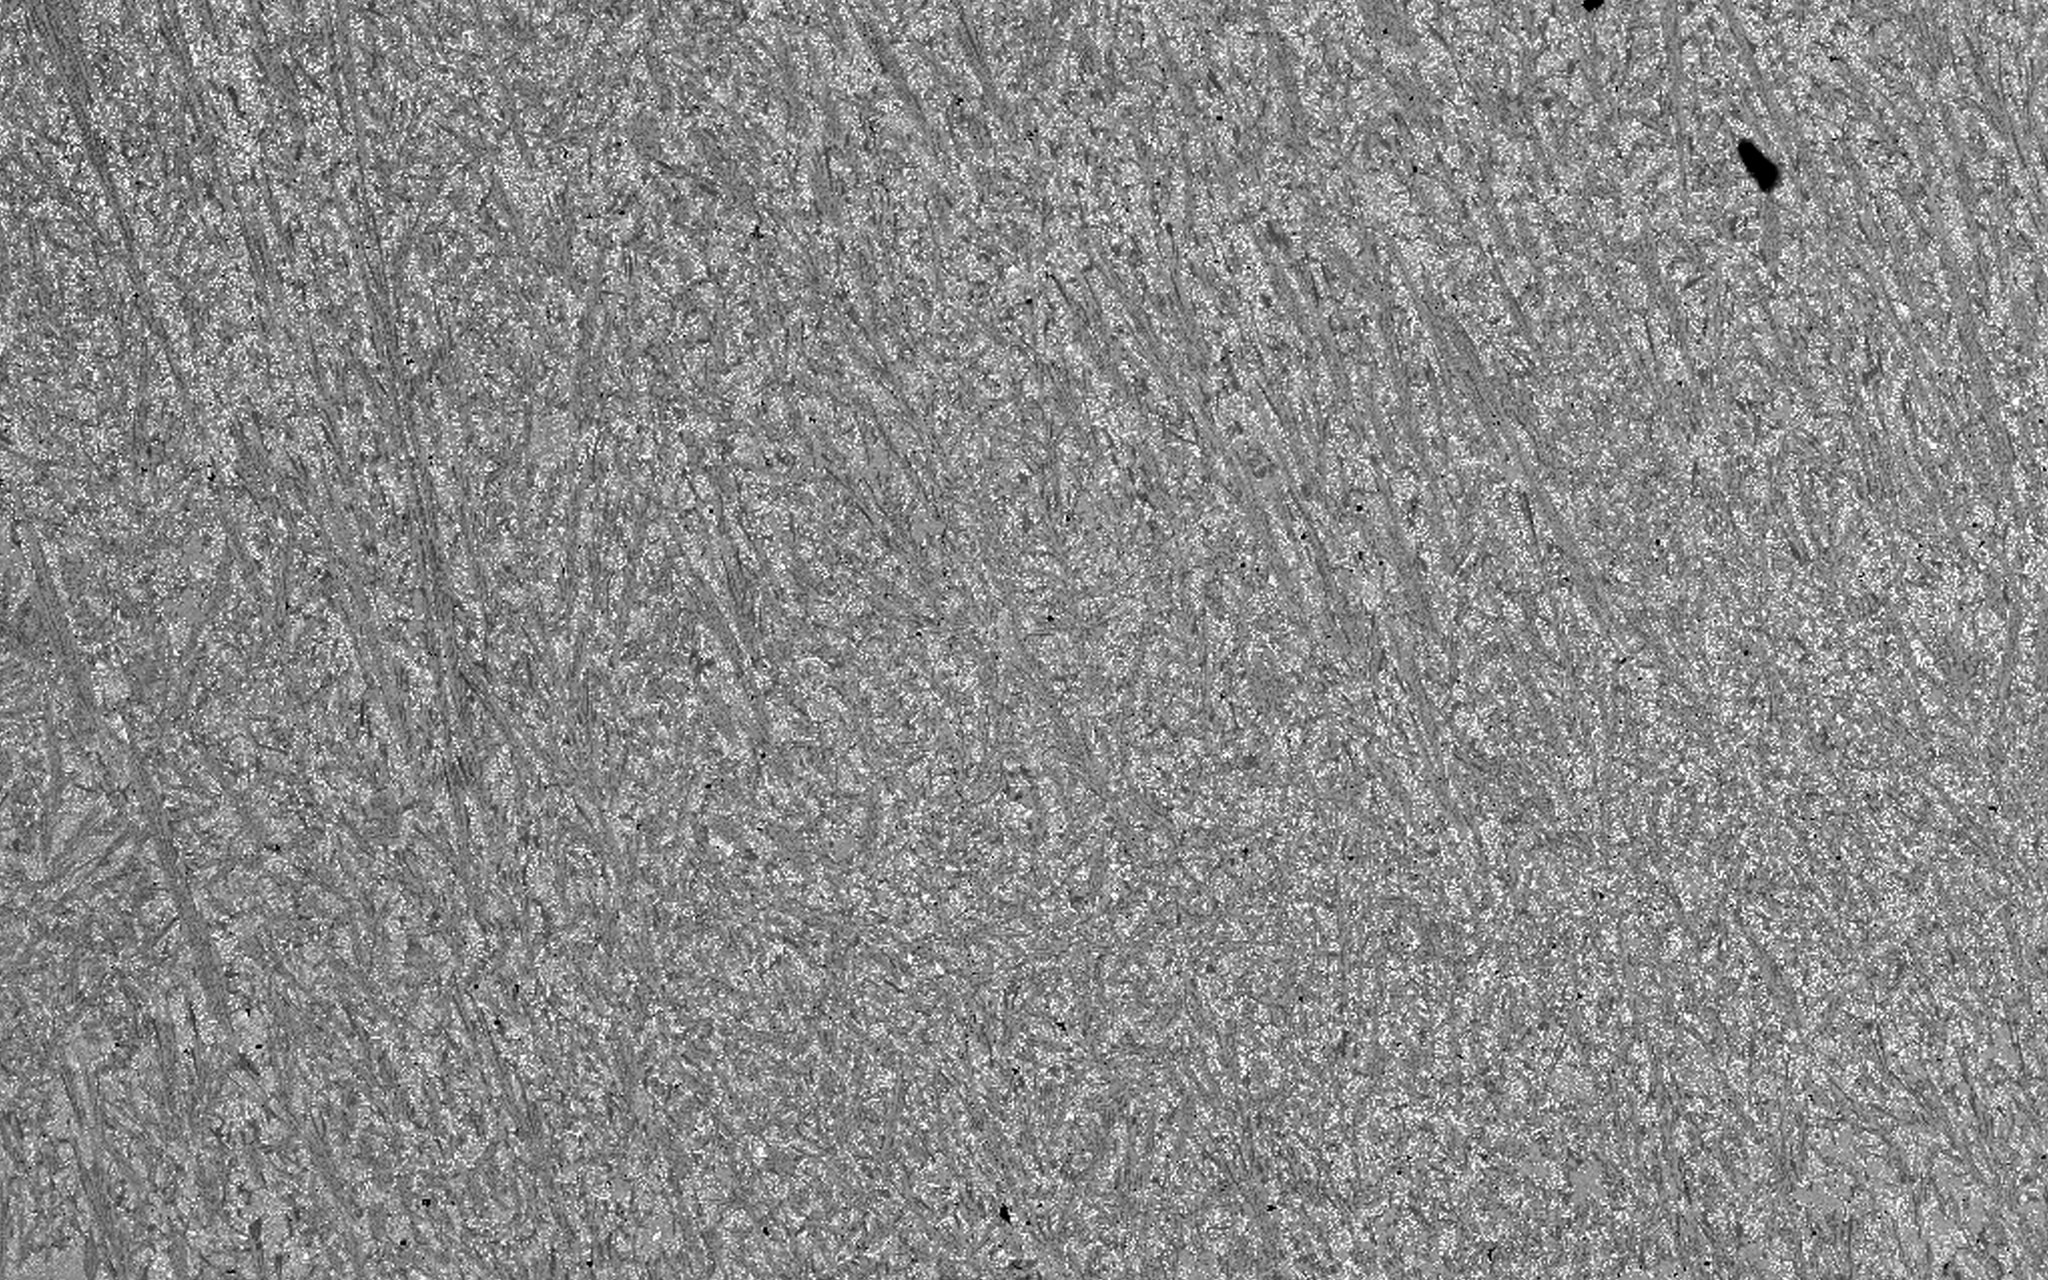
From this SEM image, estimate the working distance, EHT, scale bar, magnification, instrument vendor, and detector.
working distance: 10.77 mm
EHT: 20 kV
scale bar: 200000 nm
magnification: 0.25 K X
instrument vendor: Tescan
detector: BSE